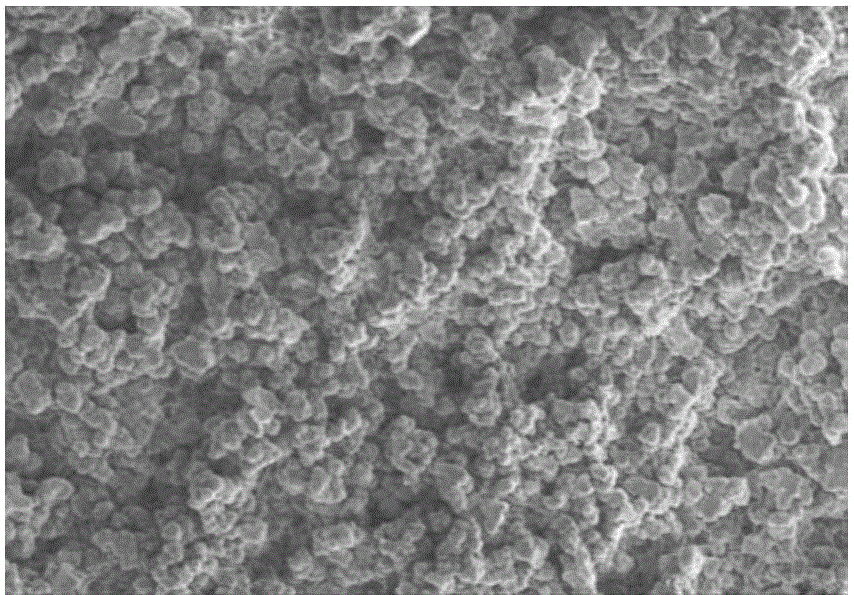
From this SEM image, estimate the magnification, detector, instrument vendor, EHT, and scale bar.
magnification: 2 K X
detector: SE(M)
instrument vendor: Hitachi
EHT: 5 kV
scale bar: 20000 nm